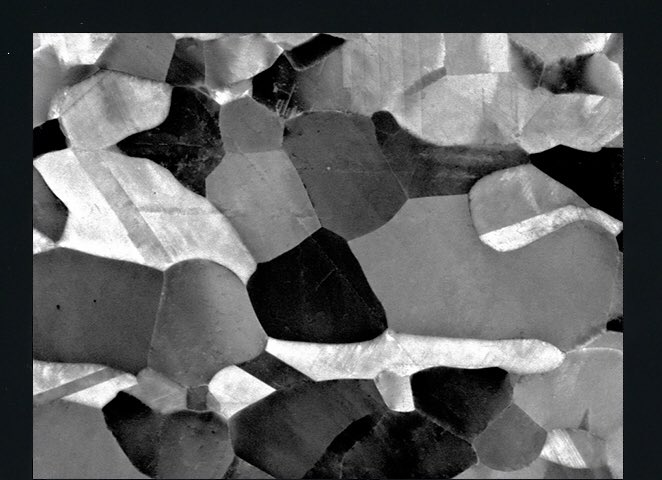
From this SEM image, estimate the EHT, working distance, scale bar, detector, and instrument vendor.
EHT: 15 kV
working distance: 3.9 mm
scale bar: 1000 nm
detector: SEI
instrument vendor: JEOL